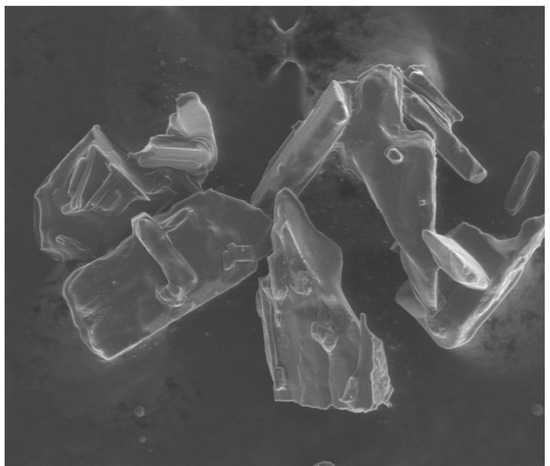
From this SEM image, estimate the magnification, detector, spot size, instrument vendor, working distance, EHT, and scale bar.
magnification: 0.3 K X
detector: LFD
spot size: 3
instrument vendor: FEI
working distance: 9.9 mm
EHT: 20 kV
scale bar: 400000 nm